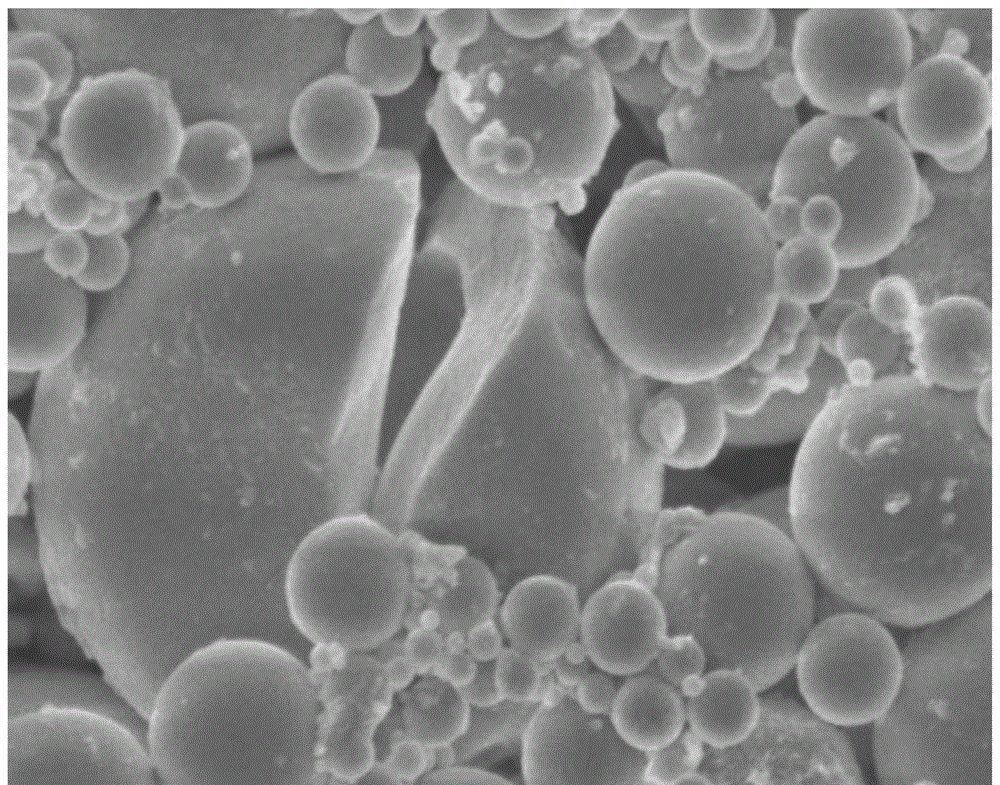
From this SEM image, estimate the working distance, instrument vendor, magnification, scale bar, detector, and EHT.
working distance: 7.8 mm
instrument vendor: JEOL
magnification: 10 K X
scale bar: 1000 nm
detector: SEI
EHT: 5 kV